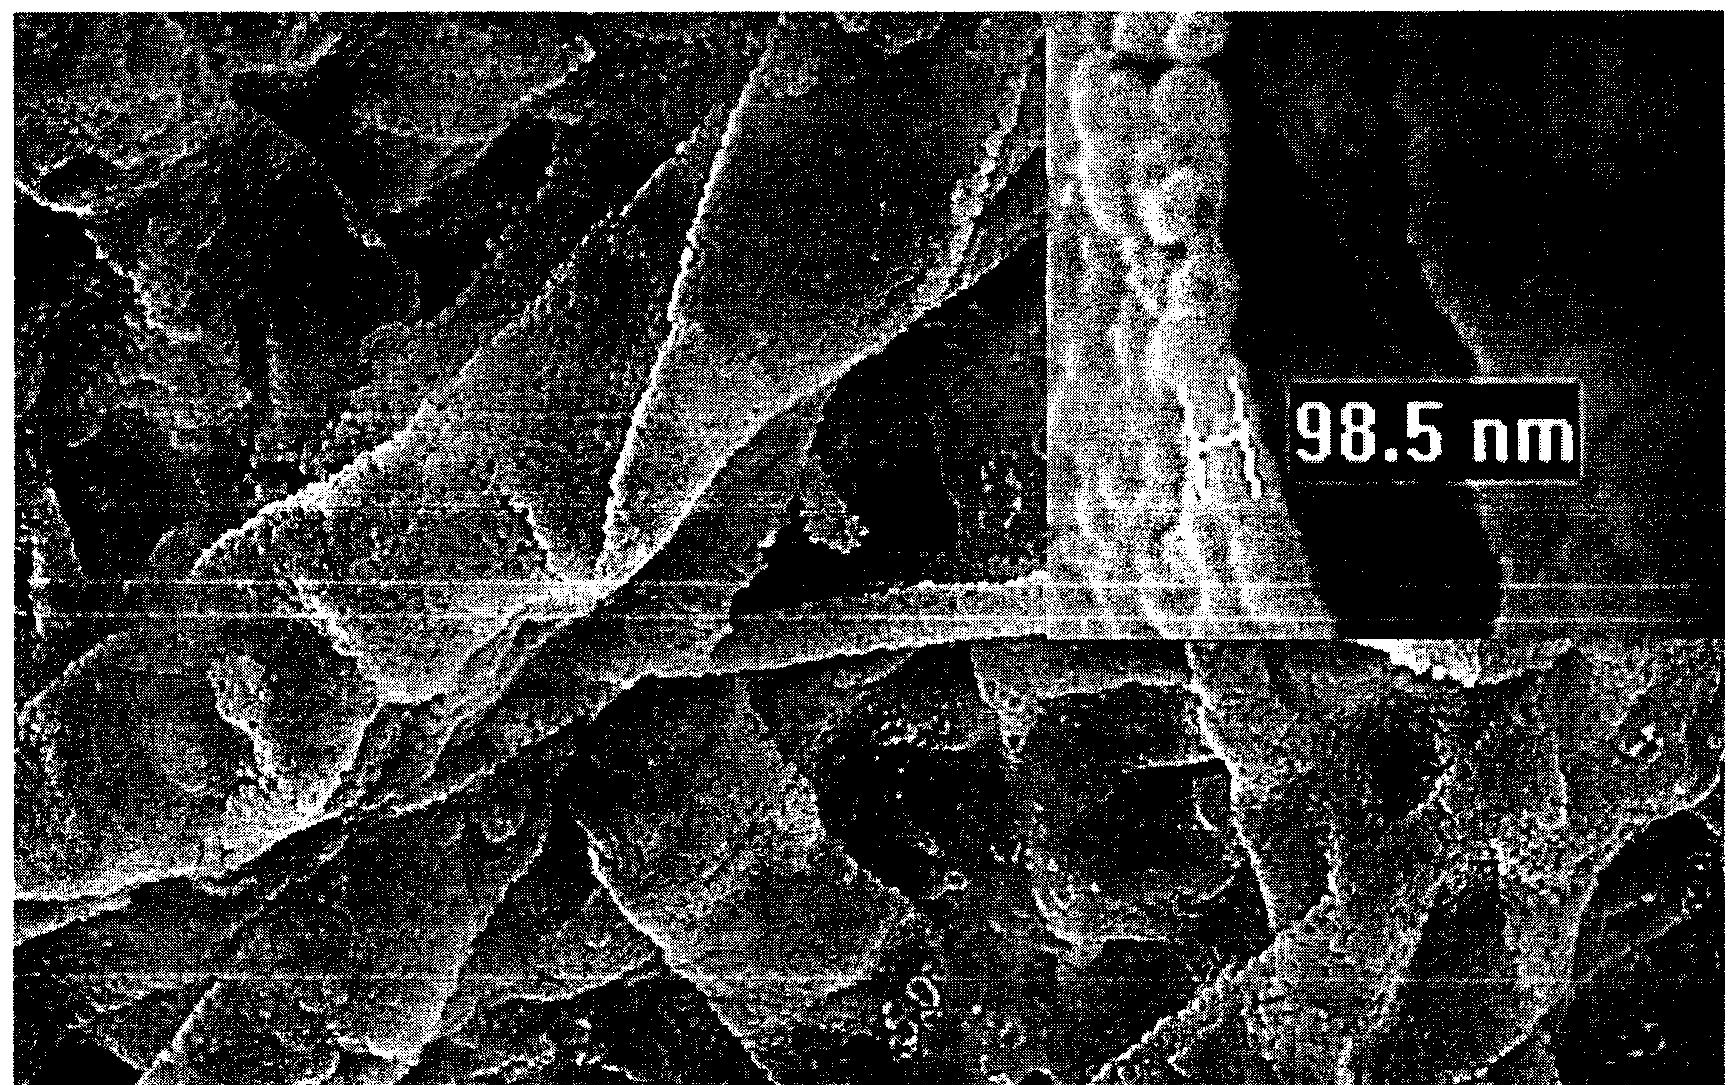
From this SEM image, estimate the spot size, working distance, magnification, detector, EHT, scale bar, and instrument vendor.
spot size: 4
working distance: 10.2 mm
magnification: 8 K X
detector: SE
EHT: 20 kV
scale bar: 2000 nm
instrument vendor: FEI